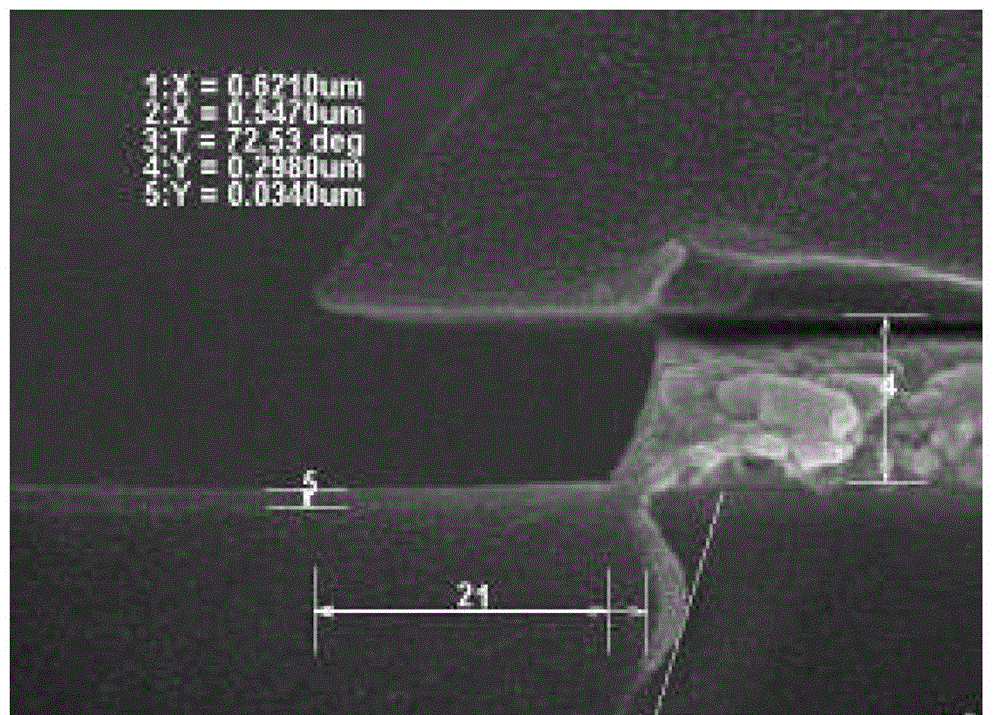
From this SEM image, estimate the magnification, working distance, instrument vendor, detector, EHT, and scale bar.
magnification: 70 K X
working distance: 12 mm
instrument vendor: Hitachi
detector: SE(U)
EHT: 15 kV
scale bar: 500 nm